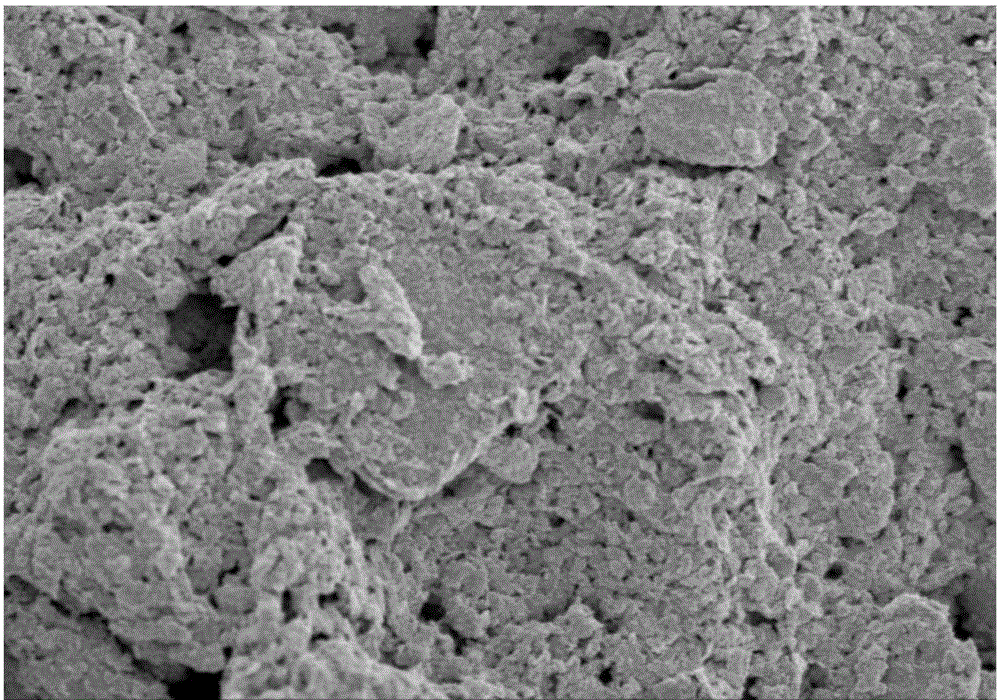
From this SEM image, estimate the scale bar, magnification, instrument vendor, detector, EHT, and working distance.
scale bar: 5000 nm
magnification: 10 K X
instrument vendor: Hitachi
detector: SE(M)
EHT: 3 kV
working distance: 9.4 mm